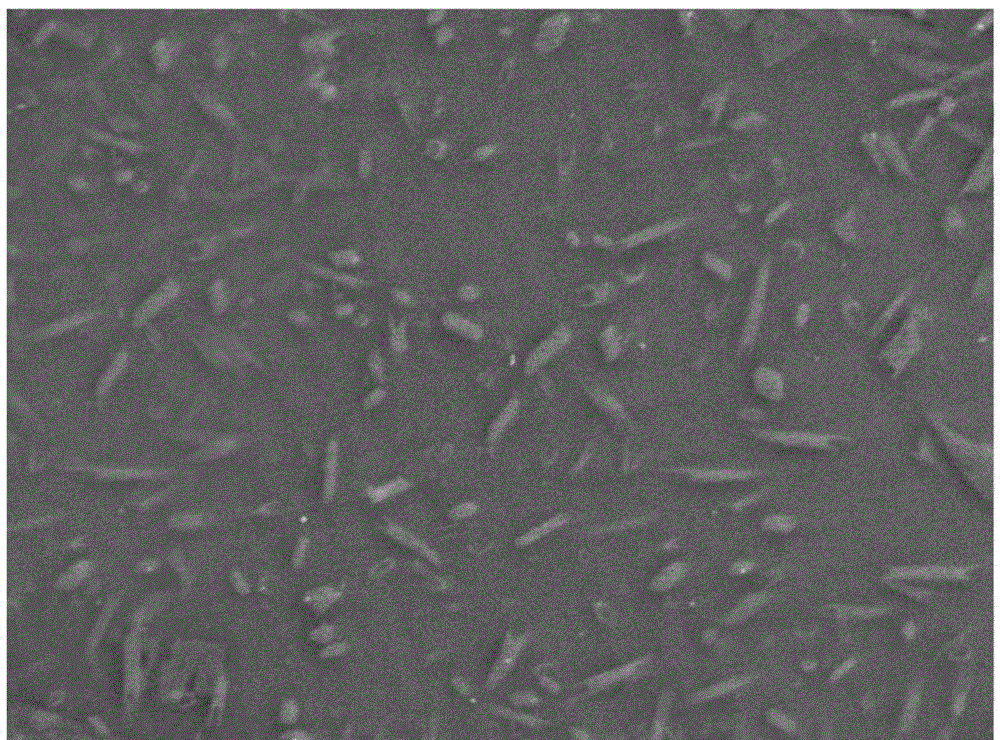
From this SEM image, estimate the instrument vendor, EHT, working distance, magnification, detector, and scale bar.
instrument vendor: JEOL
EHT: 15 kV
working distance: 10.5 mm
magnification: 2 K X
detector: SEI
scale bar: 10000 nm